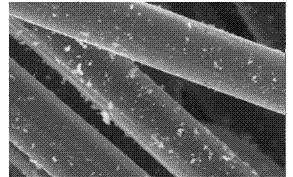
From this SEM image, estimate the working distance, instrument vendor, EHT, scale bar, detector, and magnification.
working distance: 10.2 mm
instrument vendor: FEI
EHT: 20 kV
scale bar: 10000 nm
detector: GSE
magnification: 2 K X